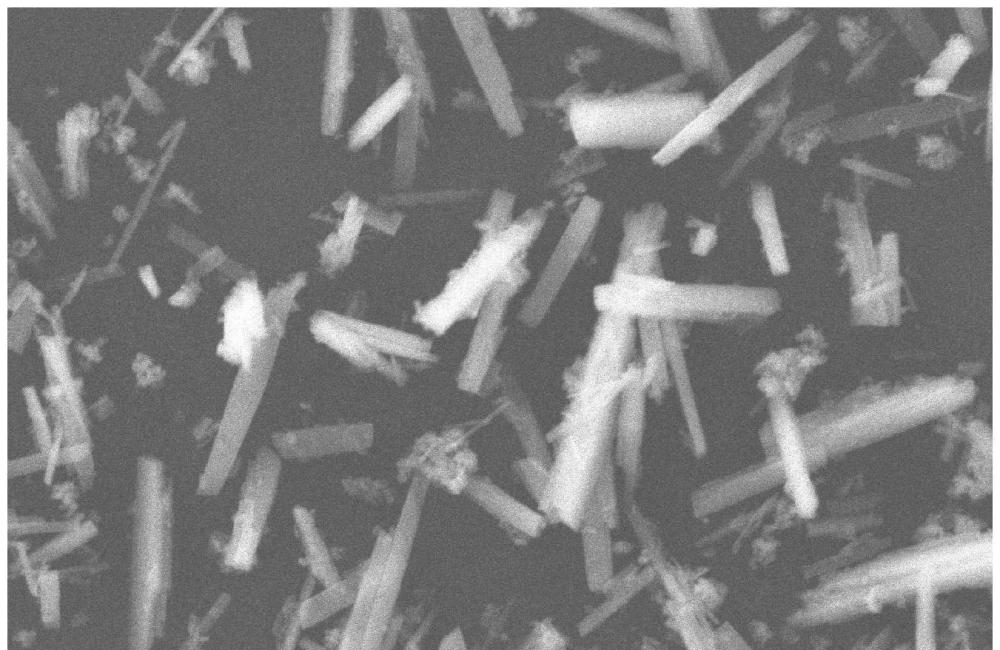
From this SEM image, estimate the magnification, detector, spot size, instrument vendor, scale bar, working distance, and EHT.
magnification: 1 K X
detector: ETD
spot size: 3.5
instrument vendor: FEI/Thermo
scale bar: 20000 nm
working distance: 11.1 mm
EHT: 30 kV